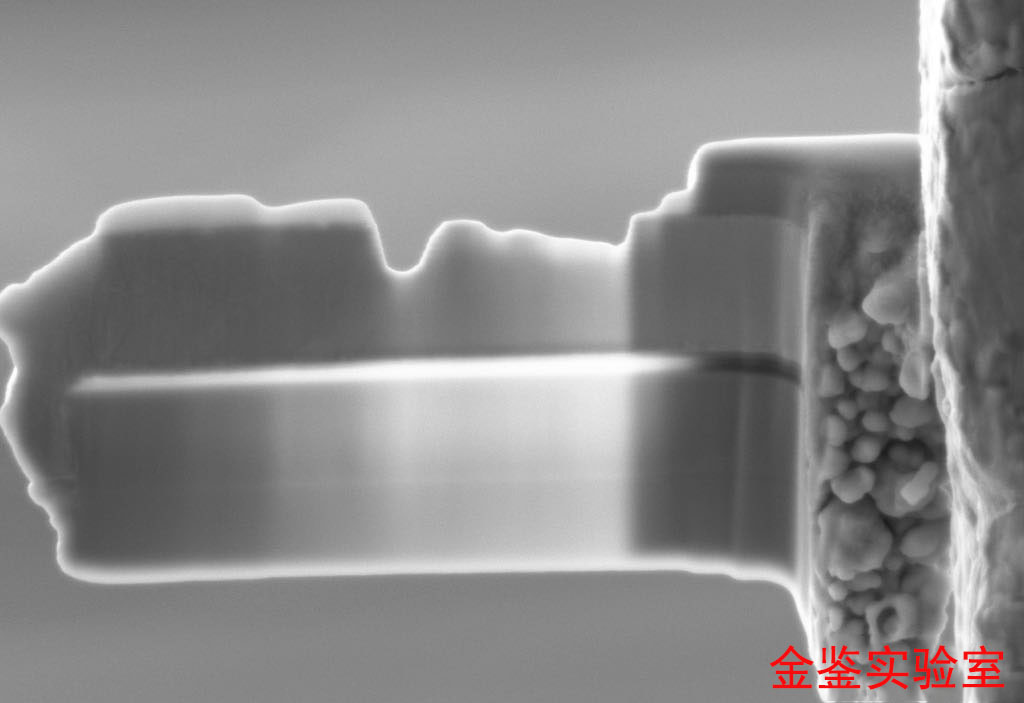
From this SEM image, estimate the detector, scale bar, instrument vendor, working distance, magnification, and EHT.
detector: SE2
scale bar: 200 nm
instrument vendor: Zeiss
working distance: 5.2 mm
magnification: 17.62 K X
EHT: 5 kV